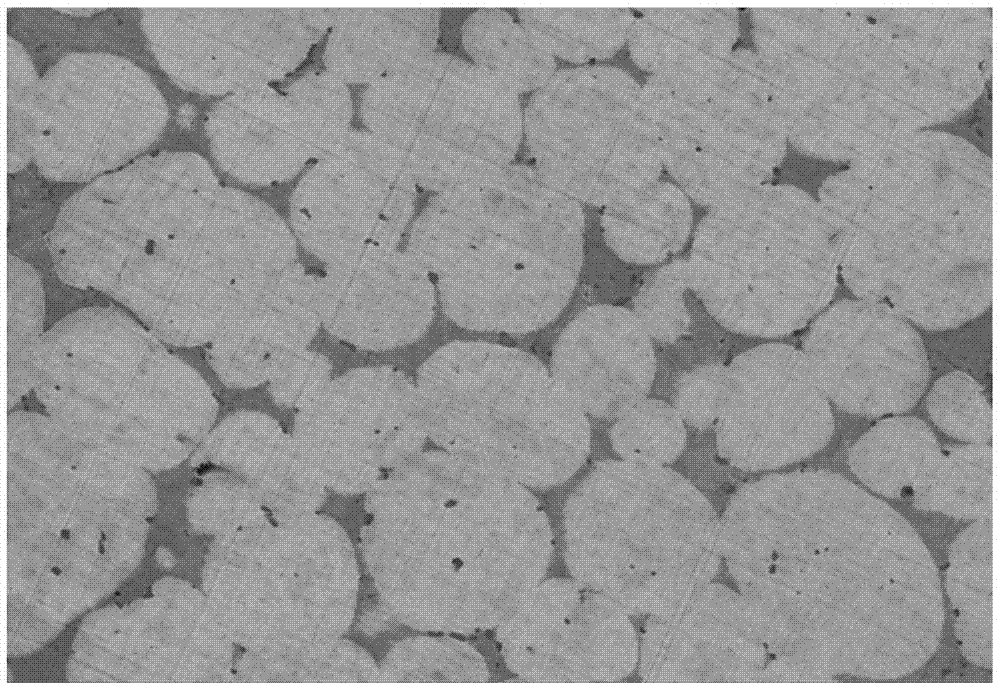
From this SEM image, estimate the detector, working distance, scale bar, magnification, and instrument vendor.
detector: SE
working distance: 12.54 mm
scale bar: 20000 nm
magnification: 2 K X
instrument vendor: Tescan